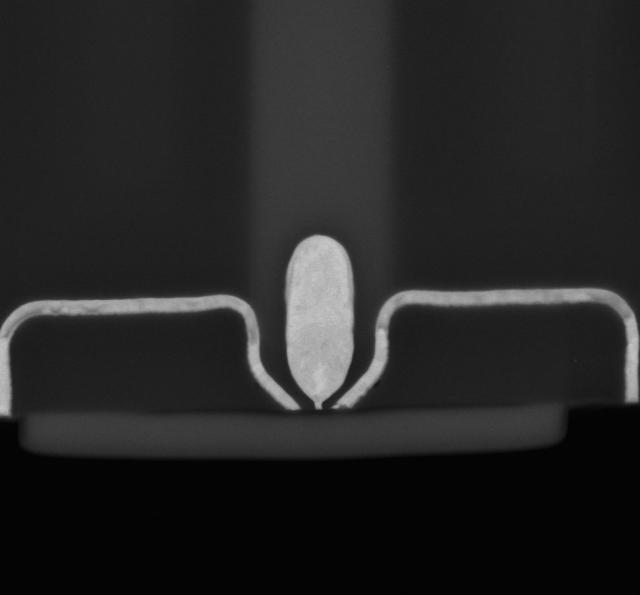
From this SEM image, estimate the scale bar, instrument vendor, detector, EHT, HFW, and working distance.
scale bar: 1000 nm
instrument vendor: FEI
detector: MD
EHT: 7 kV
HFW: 3.63 µm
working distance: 2 mm